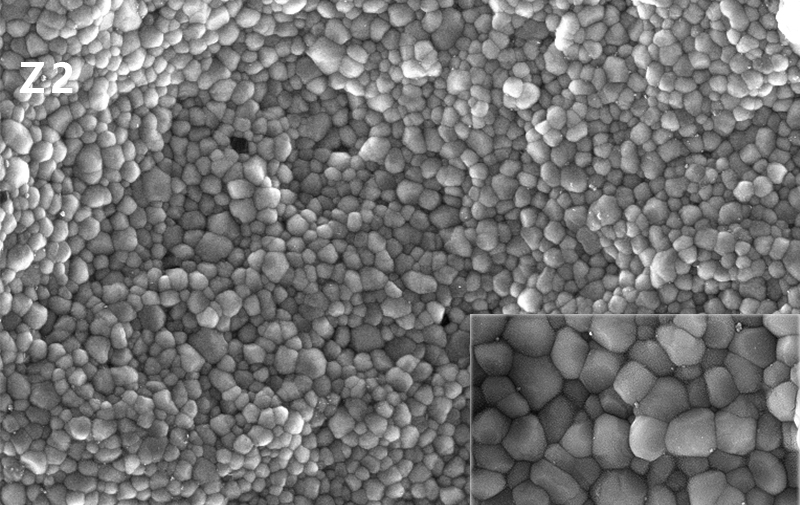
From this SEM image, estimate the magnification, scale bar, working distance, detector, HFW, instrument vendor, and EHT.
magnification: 10 K X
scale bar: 5000 nm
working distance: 5.66 mm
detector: InBeam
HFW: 21.7 µm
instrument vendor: Tescan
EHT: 10 kV